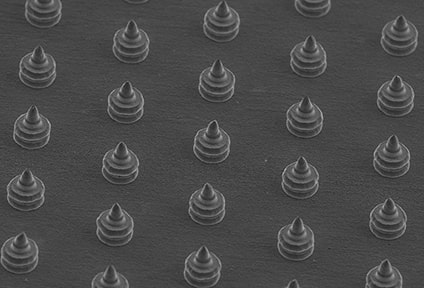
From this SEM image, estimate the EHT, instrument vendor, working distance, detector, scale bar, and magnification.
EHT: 15 kV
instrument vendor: JEOL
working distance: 11 mm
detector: SEI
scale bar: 100000 nm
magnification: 0.15 K X